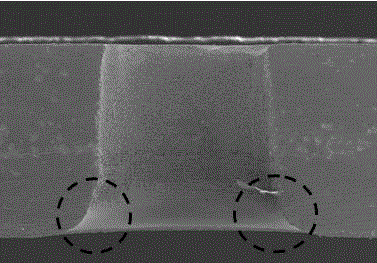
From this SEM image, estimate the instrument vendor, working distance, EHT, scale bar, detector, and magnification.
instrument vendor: Hitachi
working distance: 17.3 mm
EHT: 20 kV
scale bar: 1000000 nm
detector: SE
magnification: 0.035 K X